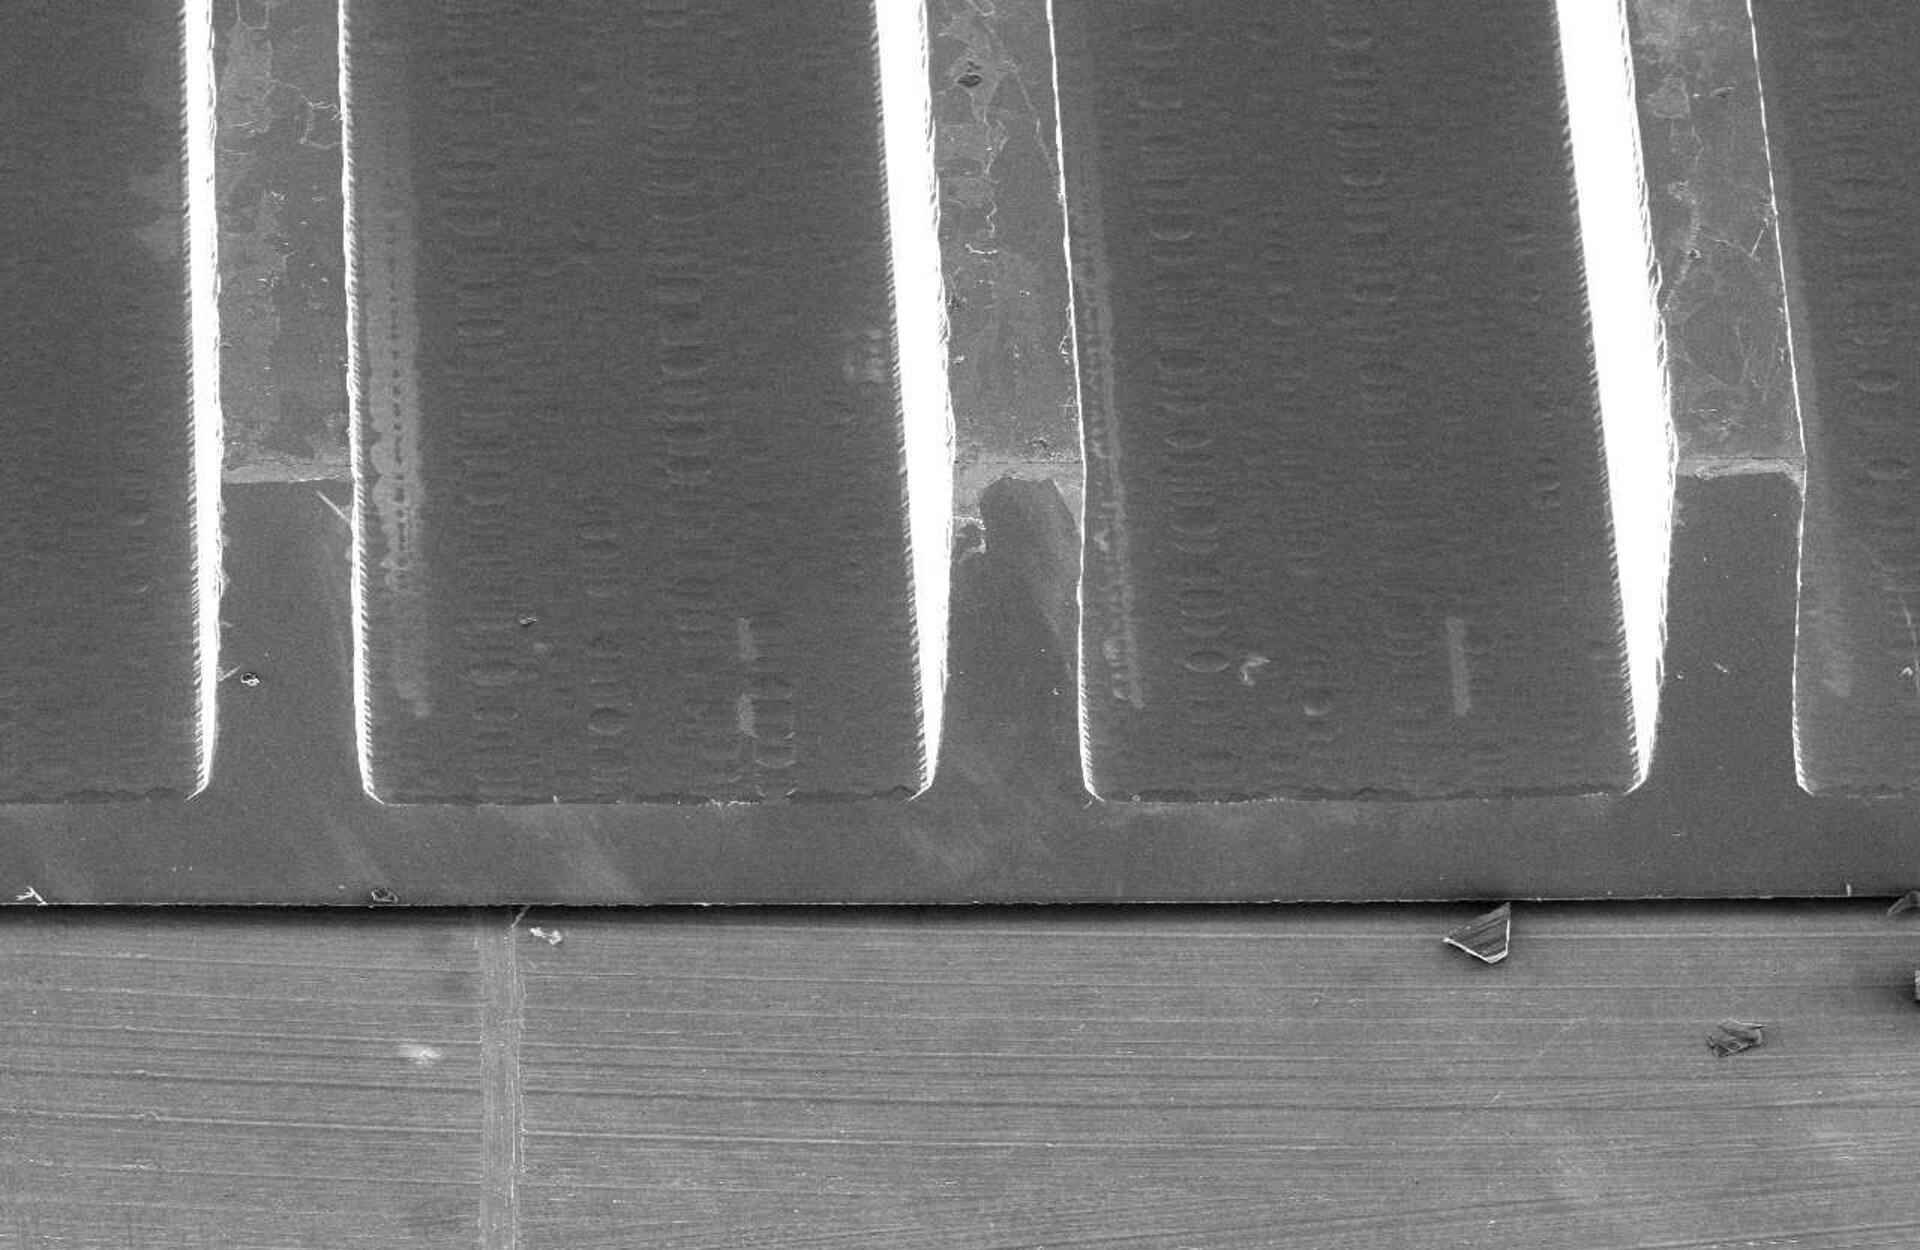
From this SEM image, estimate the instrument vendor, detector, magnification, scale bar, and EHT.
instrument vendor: JEOL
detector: SEI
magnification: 0.05 K X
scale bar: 500000 nm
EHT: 10 kV